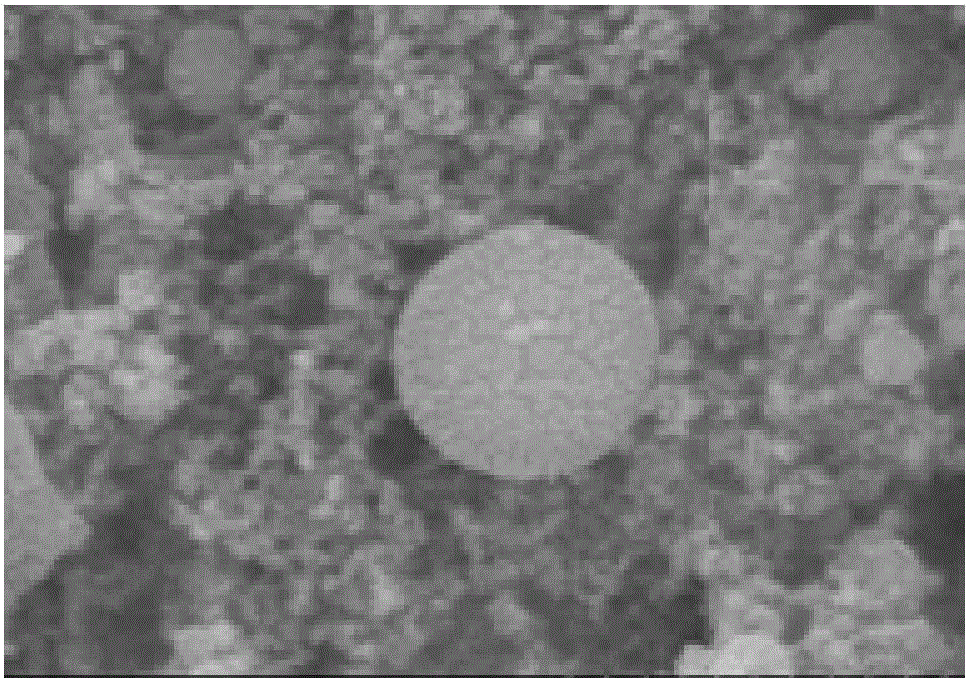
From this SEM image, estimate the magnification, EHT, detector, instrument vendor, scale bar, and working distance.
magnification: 100 K X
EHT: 15 kV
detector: SE(M)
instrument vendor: Hitachi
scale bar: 500 nm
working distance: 10 mm